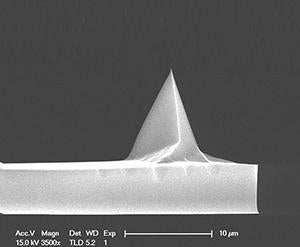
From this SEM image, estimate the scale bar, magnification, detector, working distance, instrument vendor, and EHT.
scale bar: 10000 nm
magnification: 3.5 K X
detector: TLD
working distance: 5.2 mm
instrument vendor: FEI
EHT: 15 kV